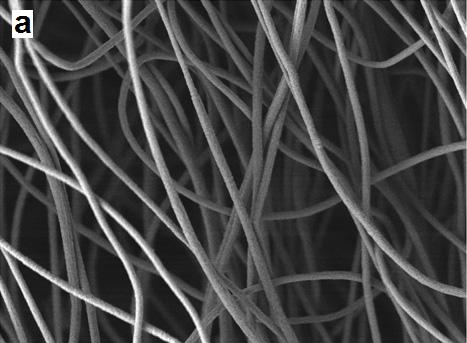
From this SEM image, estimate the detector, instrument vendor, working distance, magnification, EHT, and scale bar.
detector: LEI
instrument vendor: JEOL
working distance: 7 mm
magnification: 5 K X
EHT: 3 kV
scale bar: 1000 nm